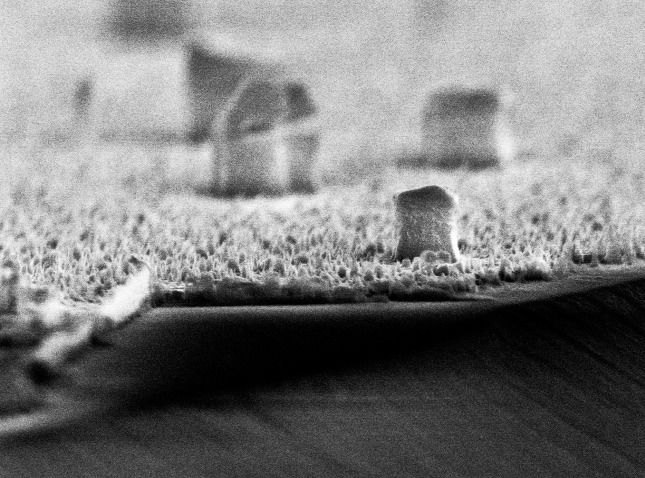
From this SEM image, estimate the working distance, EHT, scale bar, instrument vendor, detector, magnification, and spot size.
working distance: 5.6 mm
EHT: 10 kV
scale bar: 1000 nm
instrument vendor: FEI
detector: TLD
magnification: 24.008 K X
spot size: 2